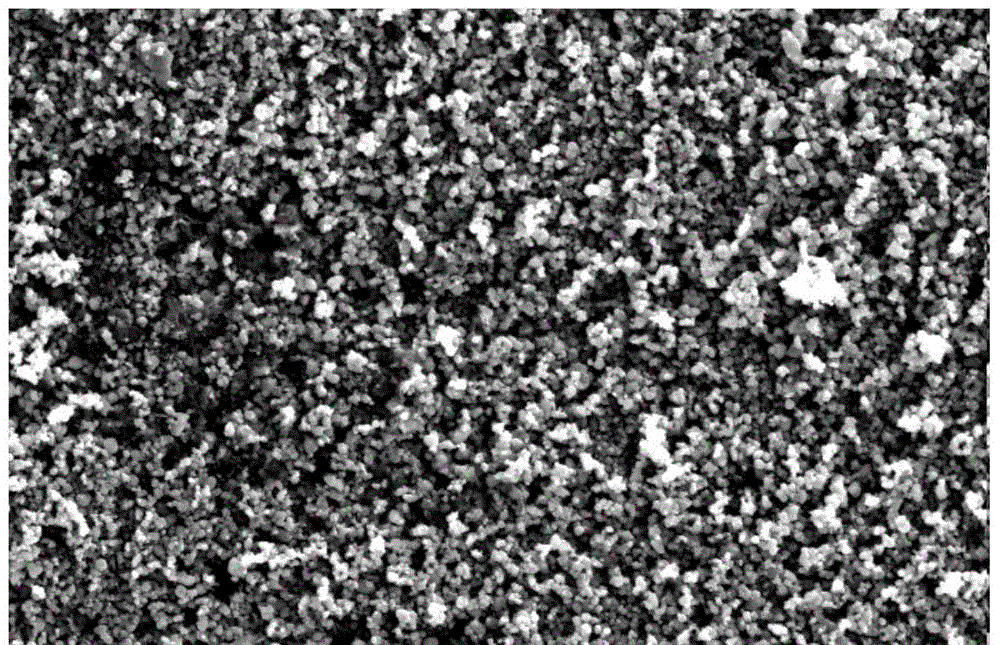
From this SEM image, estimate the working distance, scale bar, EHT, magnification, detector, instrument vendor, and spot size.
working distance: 4.4 mm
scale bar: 2000 nm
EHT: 5 kV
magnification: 20 K X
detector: SE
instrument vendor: FEI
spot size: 3